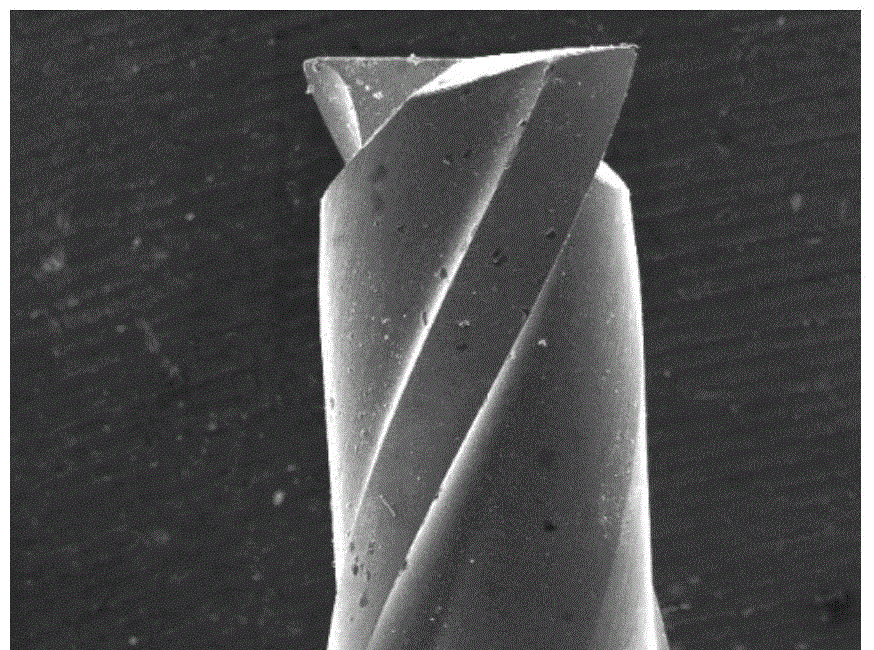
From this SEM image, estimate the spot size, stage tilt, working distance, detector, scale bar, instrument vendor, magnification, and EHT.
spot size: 5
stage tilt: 0°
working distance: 24 mm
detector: SE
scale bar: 500000 nm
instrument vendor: FEI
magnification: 0.05 K X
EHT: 20 kV